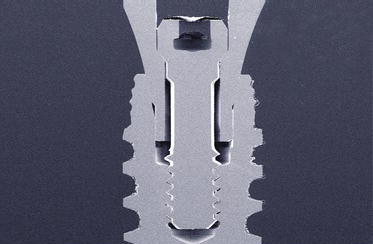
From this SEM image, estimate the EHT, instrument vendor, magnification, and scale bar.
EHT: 20 kV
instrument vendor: JEOL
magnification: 0.01 K X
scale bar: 1000000 nm